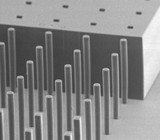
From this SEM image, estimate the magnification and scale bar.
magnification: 0.13 K X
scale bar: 200000 nm